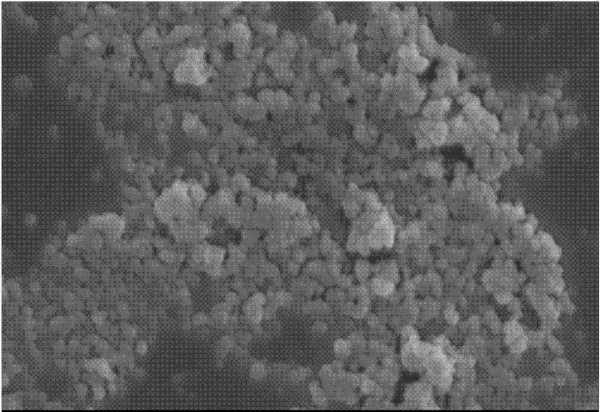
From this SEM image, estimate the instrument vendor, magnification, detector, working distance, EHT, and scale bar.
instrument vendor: Zeiss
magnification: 10 K X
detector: InLens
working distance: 8.1 mm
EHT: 15 kV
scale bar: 1000 nm